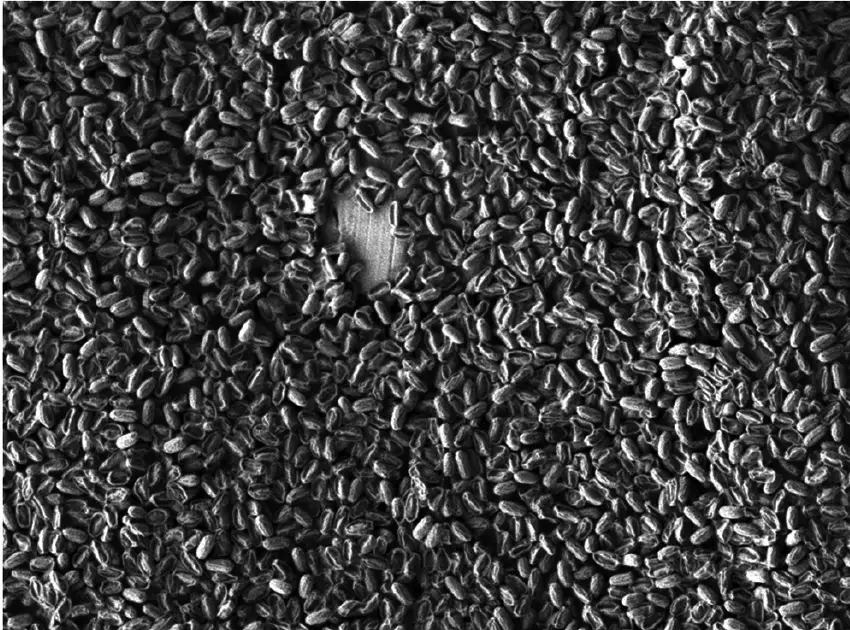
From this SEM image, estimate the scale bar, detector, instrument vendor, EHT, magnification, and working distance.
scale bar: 10000 nm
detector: LEI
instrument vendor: JEOL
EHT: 1 kV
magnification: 2.7 K X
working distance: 7.6 mm